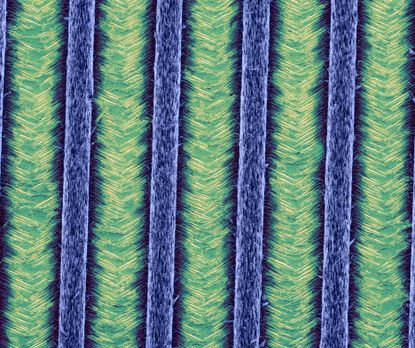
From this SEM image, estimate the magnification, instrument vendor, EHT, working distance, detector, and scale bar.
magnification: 1.25 K X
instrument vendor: FEI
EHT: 5 kV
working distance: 5.2 mm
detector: TLD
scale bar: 50000 nm